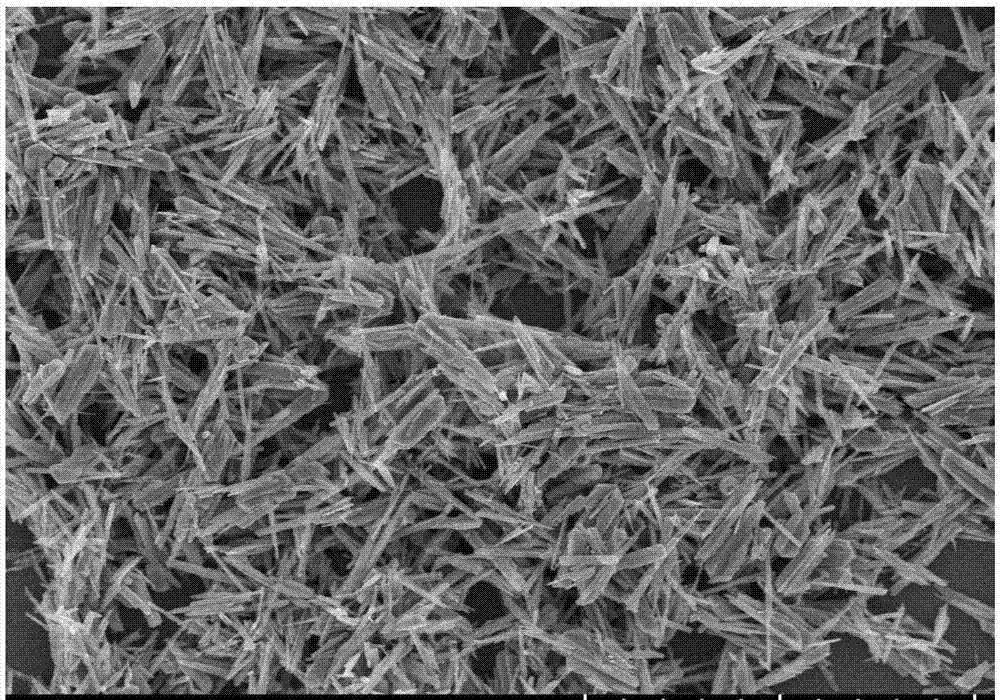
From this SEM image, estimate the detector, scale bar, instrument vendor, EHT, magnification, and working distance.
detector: SE(U)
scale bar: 5000 nm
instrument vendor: Hitachi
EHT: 5 kV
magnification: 10 K X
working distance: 8.2 mm